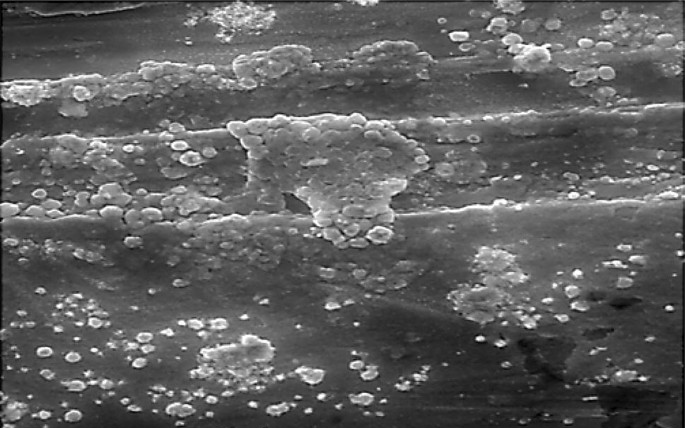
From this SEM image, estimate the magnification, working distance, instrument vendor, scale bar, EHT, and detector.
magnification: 2 K X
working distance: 13.39 mm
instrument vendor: Tescan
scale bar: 20000 nm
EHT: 20 kV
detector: SE